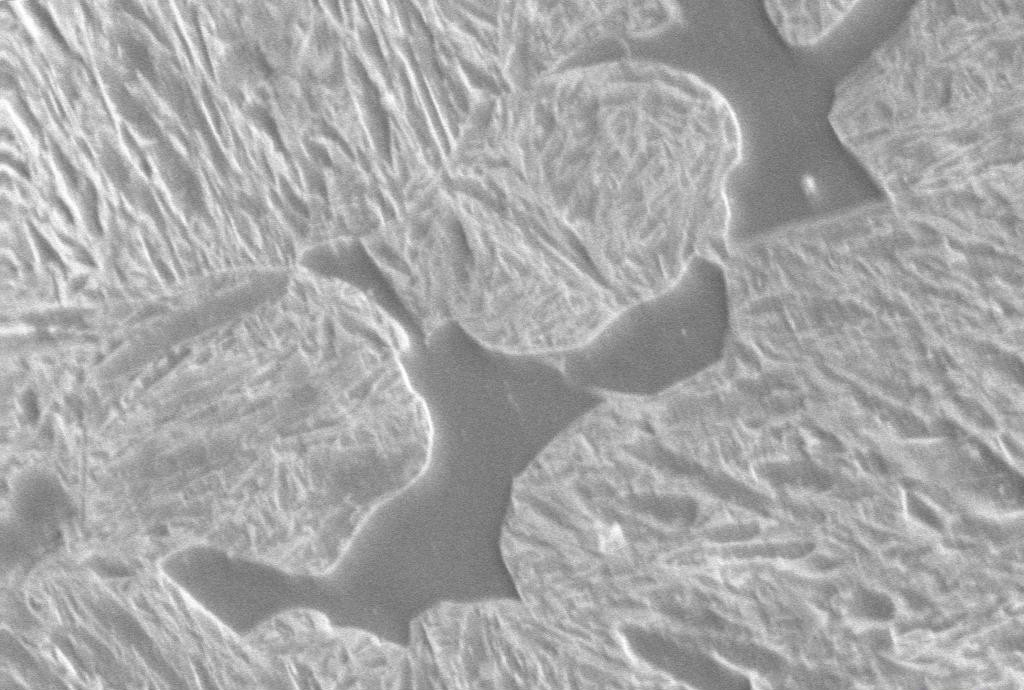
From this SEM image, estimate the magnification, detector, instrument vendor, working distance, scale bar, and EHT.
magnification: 8 K X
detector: SE1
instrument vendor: Zeiss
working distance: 10.5 mm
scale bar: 2000 nm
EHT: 10 kV